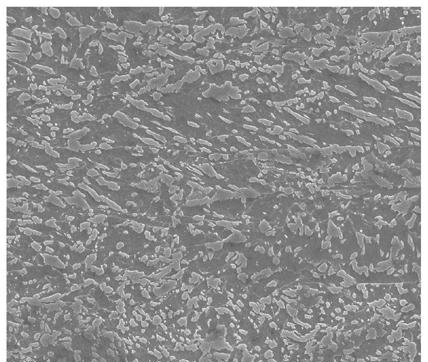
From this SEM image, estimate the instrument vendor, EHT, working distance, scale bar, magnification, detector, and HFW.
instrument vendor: FEI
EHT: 25 kV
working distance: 17.8 mm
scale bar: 30000 nm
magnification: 2 K X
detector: ETD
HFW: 74.6 µm